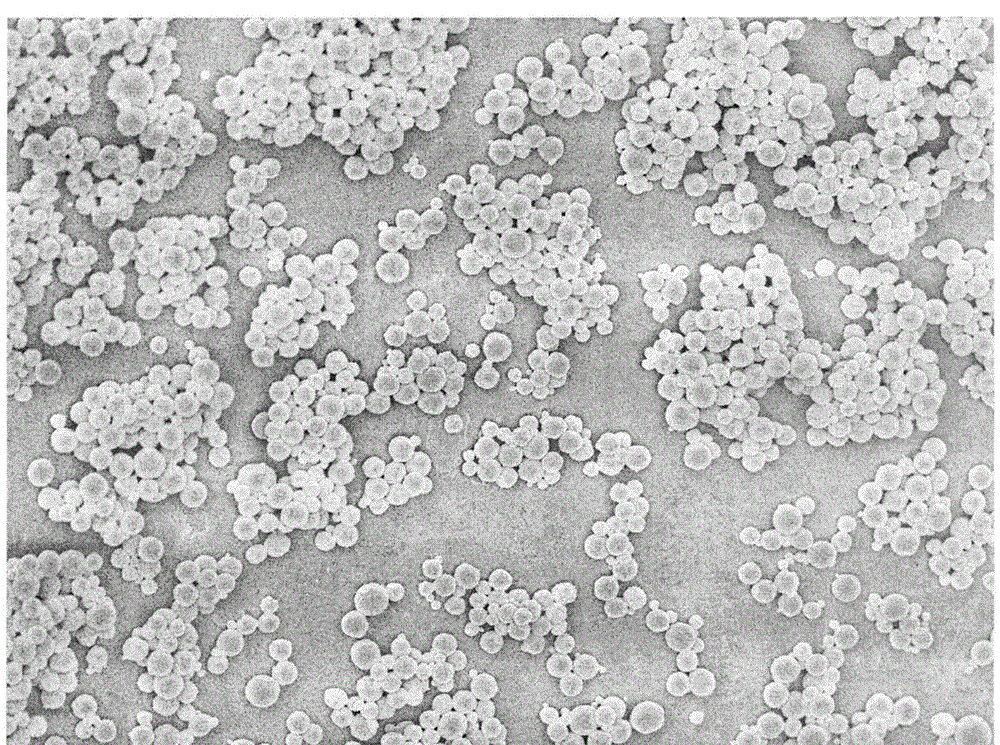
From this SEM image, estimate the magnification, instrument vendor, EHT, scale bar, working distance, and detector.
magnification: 5 K X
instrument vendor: JEOL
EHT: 5 kV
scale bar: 1000 nm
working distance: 7 mm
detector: SEI